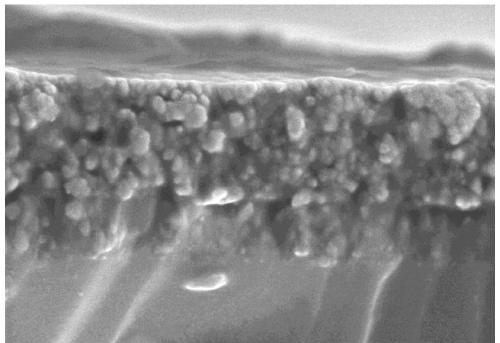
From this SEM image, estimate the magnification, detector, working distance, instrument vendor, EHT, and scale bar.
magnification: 40 K X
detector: SE(M)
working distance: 9.6 mm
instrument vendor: Hitachi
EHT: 3 kV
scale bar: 1000 nm